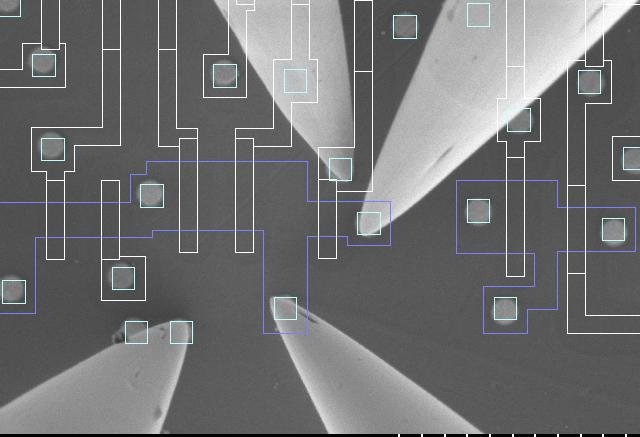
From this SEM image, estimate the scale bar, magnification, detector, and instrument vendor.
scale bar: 3000 nm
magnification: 15 K X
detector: SE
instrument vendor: Hitachi